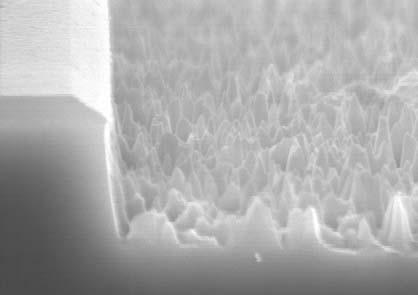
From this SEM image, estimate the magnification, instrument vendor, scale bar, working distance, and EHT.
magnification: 30 K X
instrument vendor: Hitachi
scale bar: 1000 nm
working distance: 11.6 mm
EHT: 15 kV